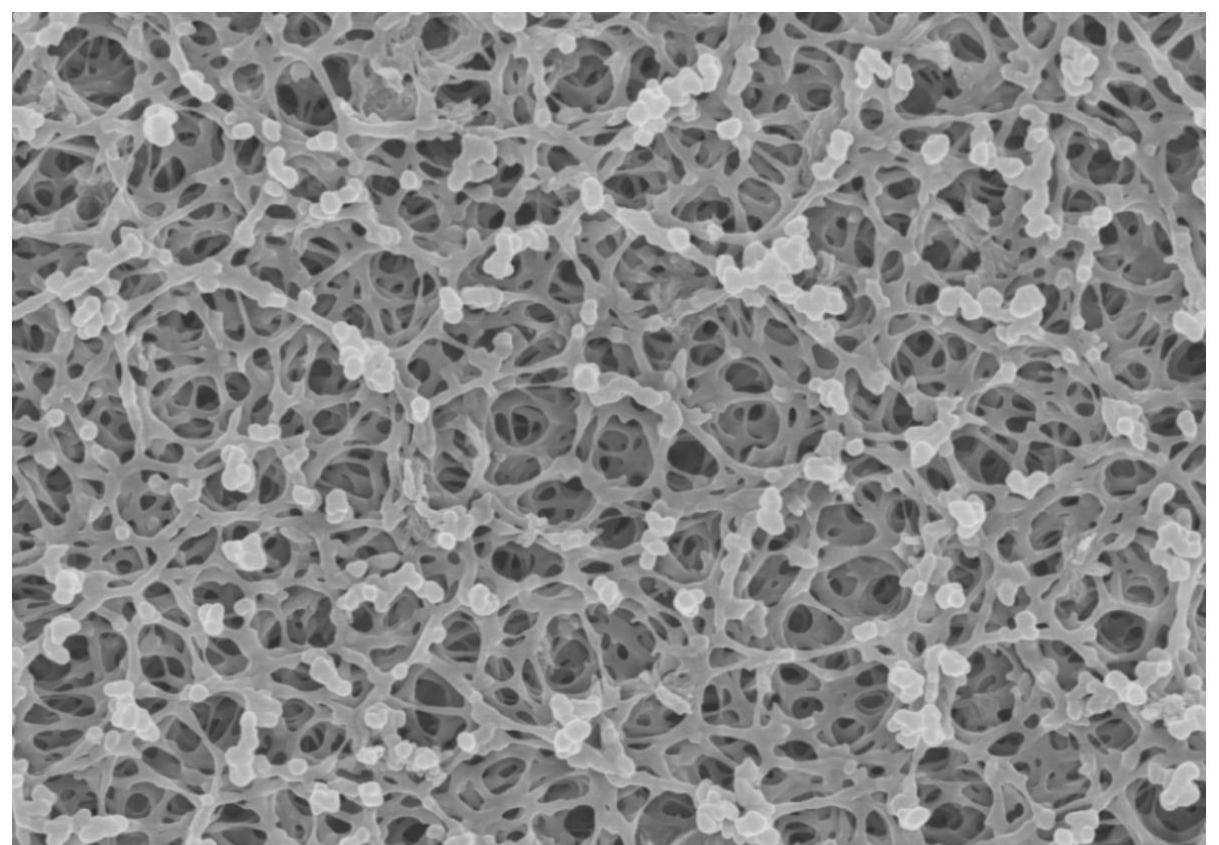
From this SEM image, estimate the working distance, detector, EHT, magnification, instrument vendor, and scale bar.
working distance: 8.8 mm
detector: SE(M)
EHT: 5 kV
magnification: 10 K X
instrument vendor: Hitachi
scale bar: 5000 nm